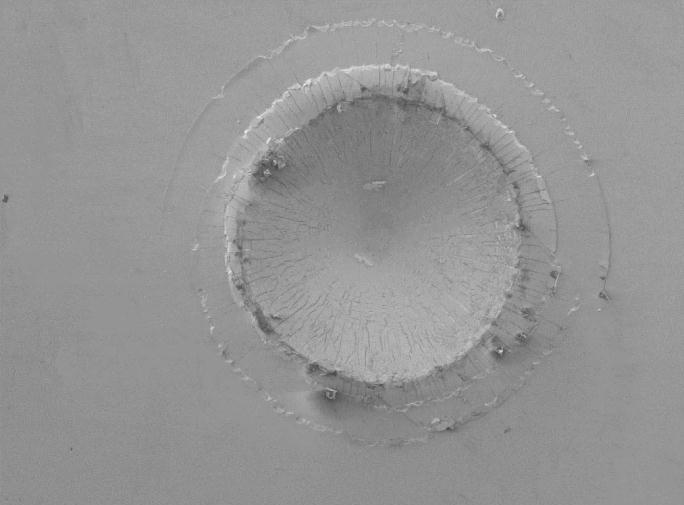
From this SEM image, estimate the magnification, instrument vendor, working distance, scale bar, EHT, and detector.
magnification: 0.05 K X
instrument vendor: JEOL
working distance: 7 mm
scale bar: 100000 nm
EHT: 5 kV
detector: SEI